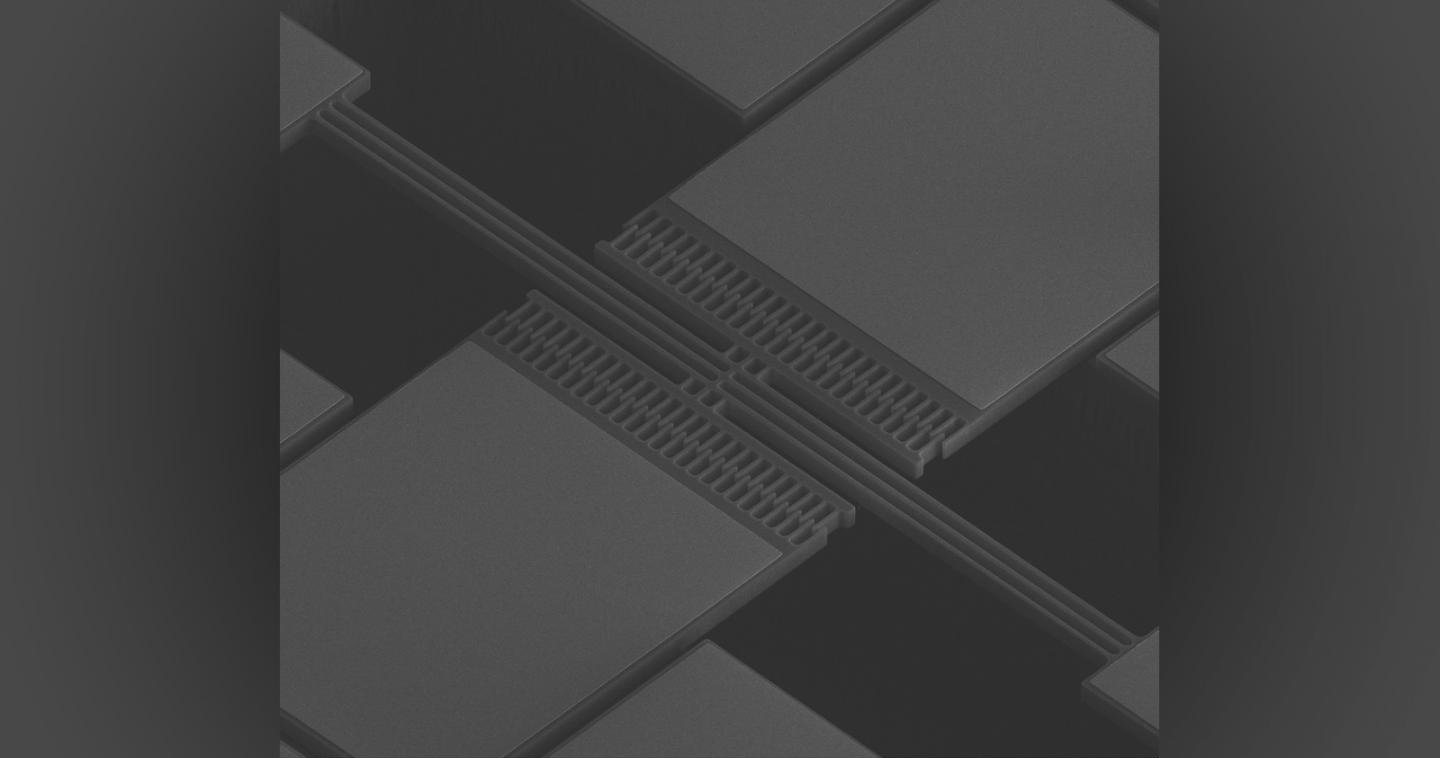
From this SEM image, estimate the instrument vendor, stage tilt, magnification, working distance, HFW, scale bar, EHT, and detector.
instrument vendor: FEI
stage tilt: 45°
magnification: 0.325 K X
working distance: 5.4 mm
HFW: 394 µm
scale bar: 100000 nm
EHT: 15 kV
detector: ETD